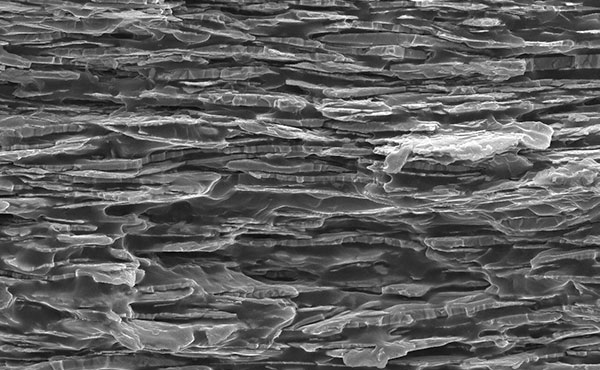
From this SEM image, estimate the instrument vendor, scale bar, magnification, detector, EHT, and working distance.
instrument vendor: JEOL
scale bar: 10000 nm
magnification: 1 K X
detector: SEI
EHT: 15 kV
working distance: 14.9 mm